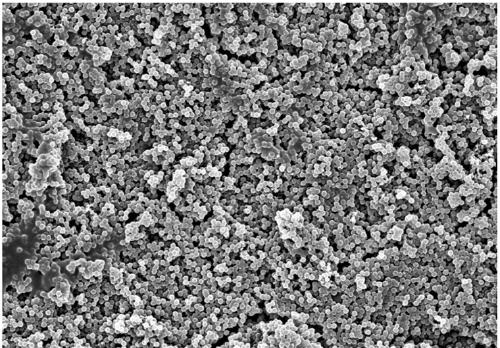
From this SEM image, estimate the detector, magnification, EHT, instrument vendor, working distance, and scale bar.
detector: SE(U)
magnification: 5 K X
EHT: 5 kV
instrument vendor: Hitachi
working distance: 7.4 mm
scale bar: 10000 nm